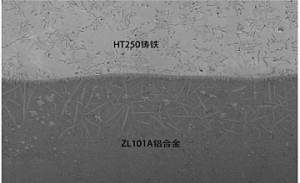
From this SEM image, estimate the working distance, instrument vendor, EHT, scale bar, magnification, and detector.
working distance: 15.4 mm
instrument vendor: Hitachi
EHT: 15 kV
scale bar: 200000 nm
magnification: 0.2 K X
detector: SE(M)